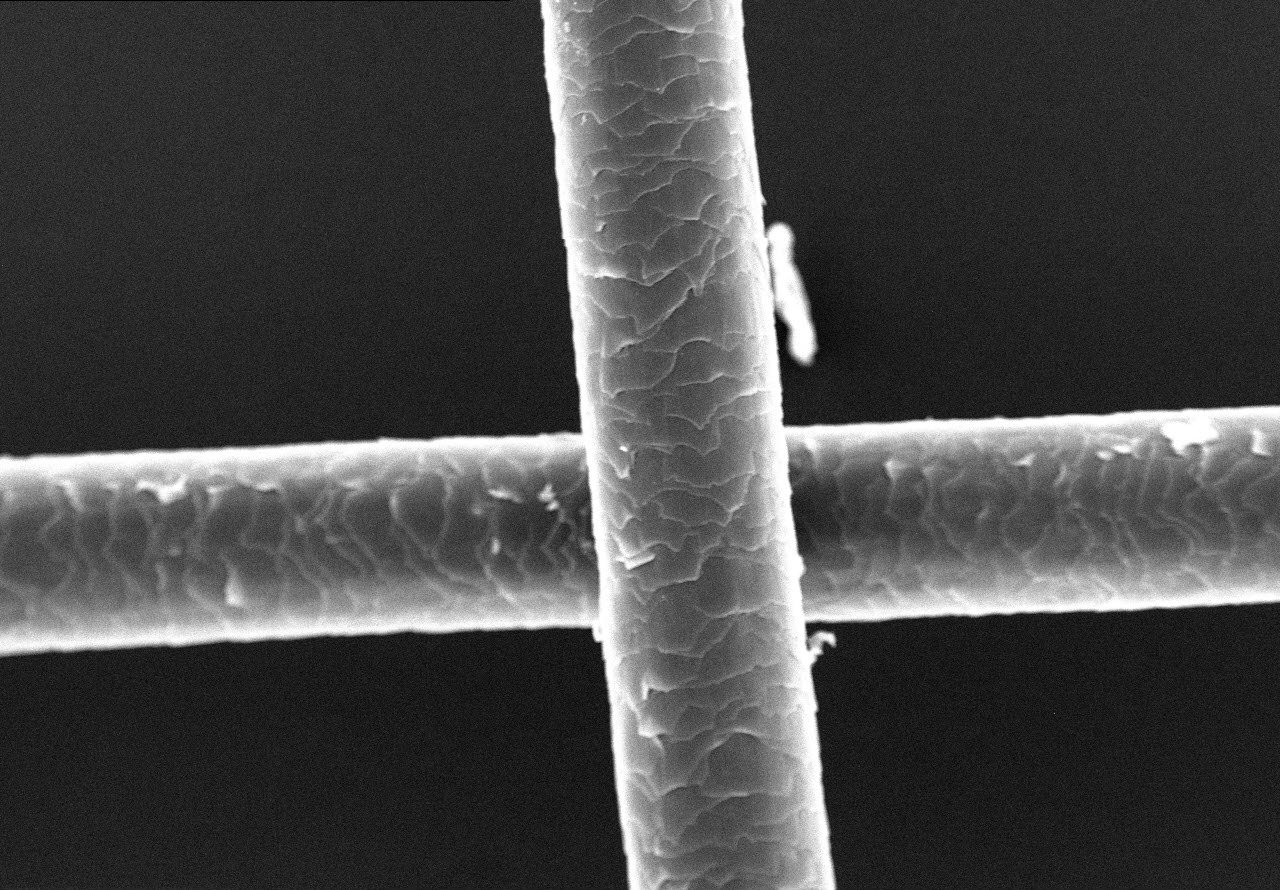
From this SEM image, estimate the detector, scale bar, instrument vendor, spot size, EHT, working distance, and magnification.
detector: SEI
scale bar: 100000 nm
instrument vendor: other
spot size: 10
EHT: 20 kV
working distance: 11.7 mm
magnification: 0.495 K X